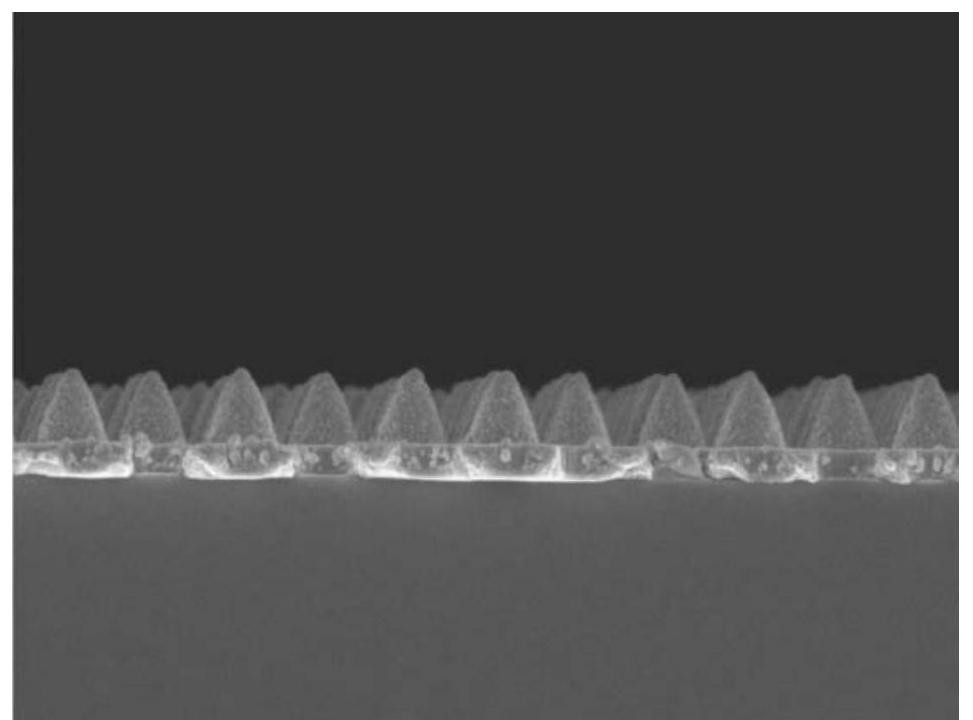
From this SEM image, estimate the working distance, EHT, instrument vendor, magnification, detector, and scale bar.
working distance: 7.6 mm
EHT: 3 kV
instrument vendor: JEOL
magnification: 25 K X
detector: SEI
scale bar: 1000 nm